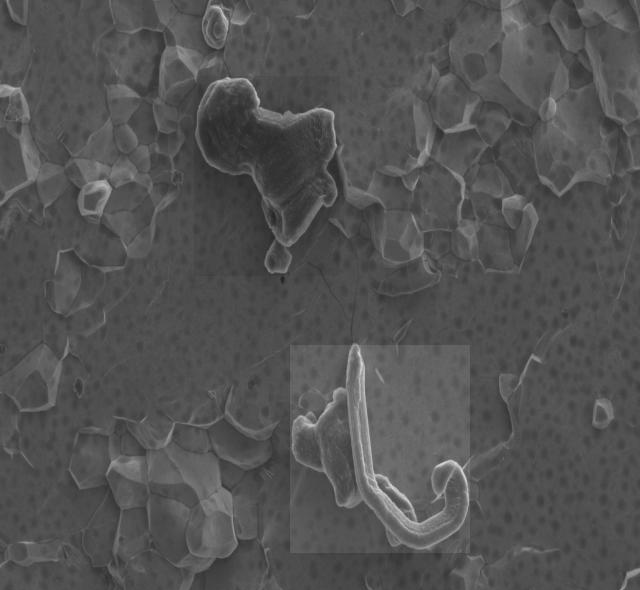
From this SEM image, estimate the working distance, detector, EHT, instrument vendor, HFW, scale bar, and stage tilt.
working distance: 4.5 mm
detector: ETD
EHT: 2 kV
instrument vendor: FEI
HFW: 27.6 µm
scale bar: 10000 nm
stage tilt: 0°